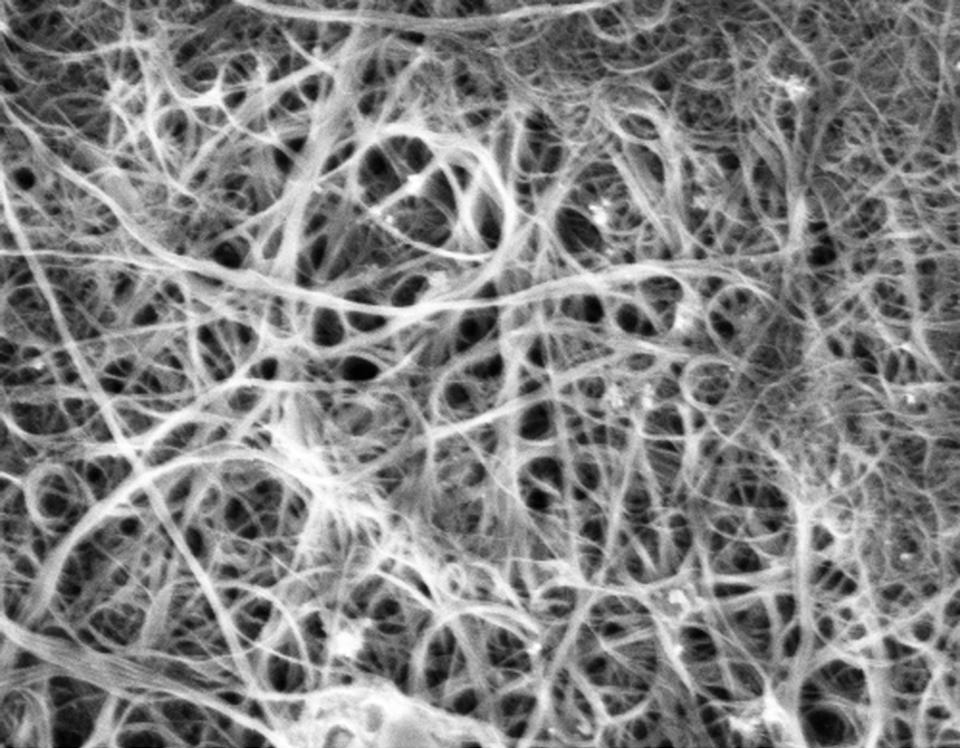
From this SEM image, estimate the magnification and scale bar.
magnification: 25 K X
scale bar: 1000 nm